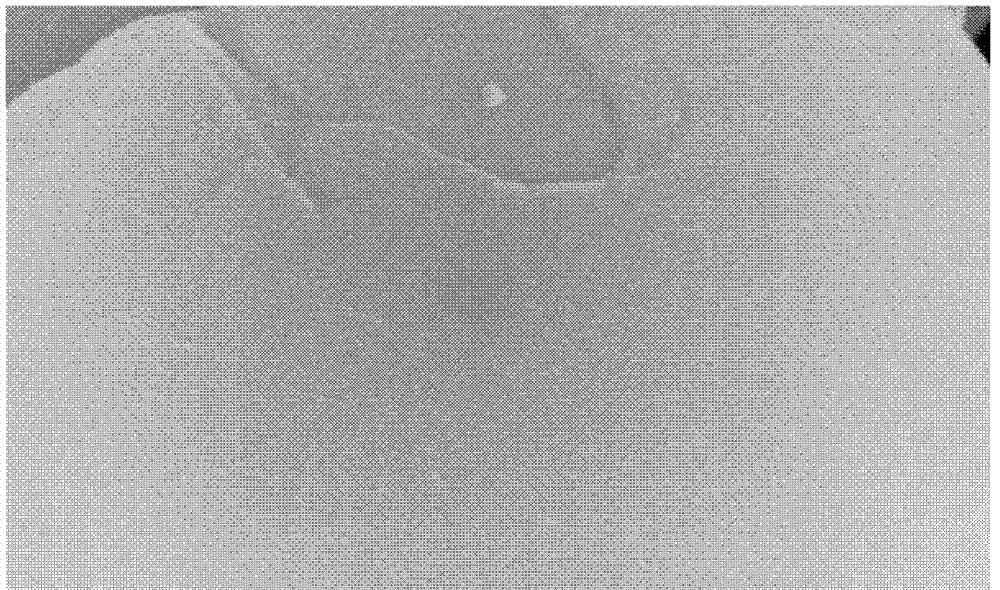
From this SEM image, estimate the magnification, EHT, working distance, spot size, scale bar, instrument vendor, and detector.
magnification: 40 K X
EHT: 15 kV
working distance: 6.9 mm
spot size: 3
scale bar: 500 nm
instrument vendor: FEI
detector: SE